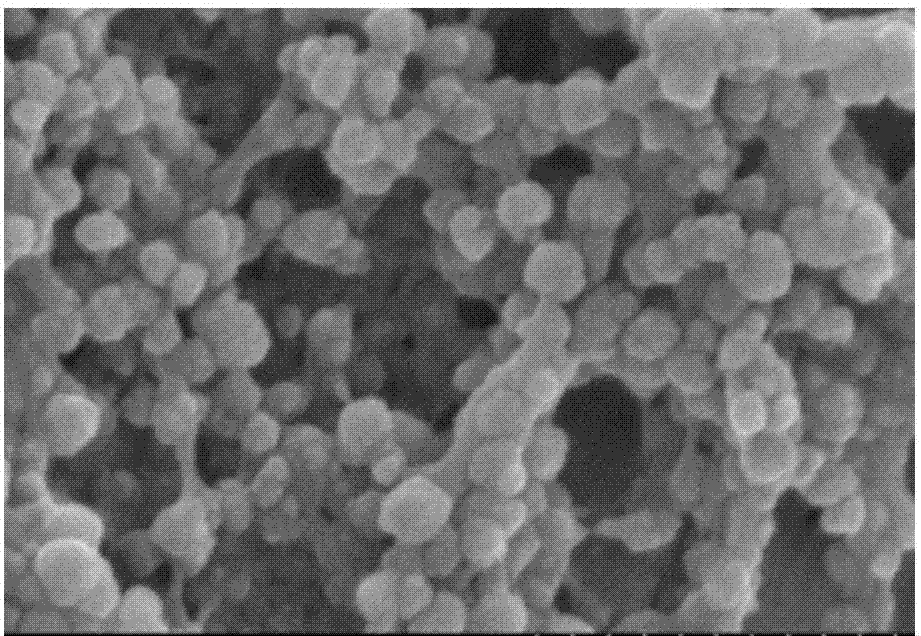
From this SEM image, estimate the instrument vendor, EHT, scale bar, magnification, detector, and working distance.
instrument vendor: Hitachi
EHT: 3 kV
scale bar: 500 nm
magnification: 100 K X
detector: SE(M)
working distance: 9.4 mm